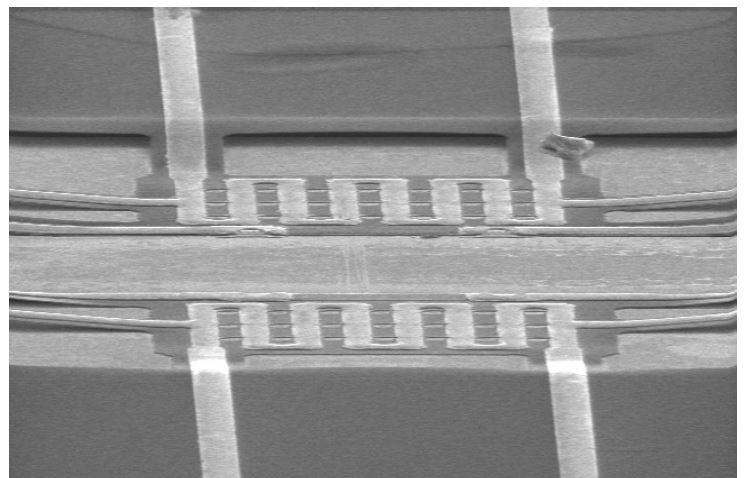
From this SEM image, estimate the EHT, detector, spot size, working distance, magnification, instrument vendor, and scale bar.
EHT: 10 kV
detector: SE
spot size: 3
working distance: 18.2 mm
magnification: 1.5 K X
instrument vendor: FEI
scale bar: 20000 nm